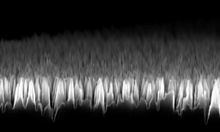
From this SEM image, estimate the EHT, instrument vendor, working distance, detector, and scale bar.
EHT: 10 kV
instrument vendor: Zeiss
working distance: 5 mm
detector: InLens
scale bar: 1000 nm